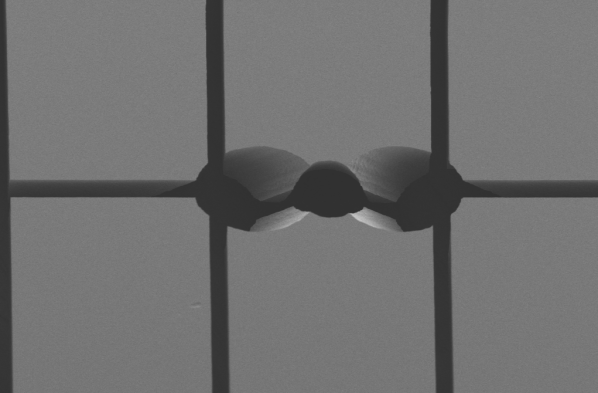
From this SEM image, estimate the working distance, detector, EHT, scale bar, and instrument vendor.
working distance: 5.7 mm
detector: SE2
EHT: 2 kV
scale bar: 100000 nm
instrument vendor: Zeiss